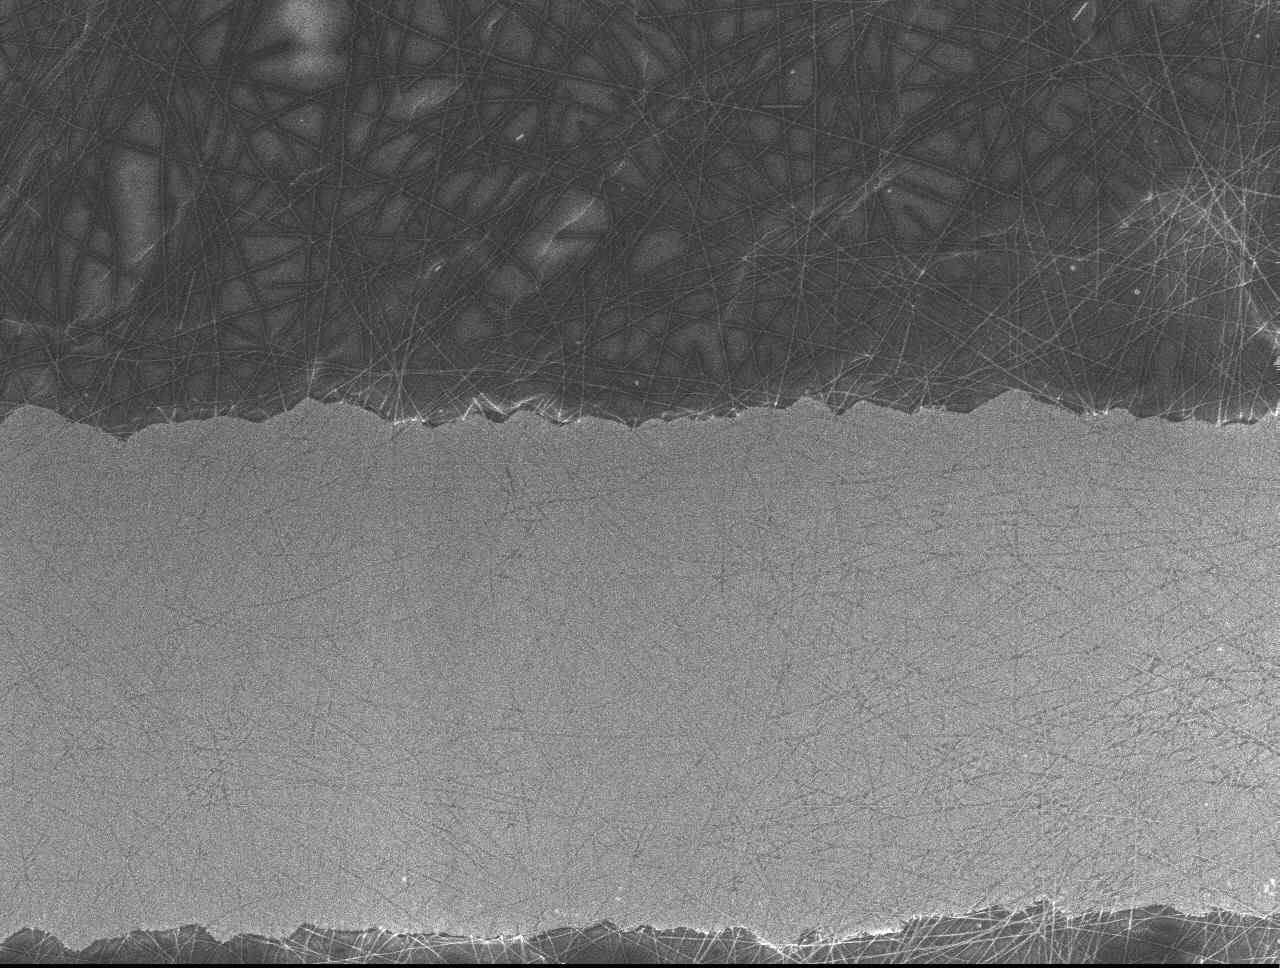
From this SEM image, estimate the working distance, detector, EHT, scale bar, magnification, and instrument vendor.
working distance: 7 mm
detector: SEI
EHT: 5 kV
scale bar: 1000 nm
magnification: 4.3 K X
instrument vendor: JEOL